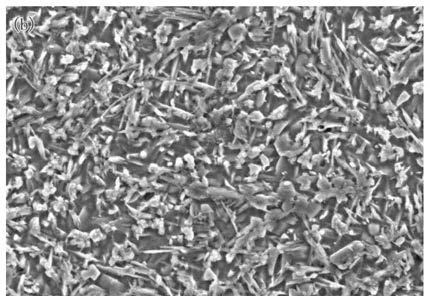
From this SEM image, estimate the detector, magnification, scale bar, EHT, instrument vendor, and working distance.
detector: SE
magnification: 2 K X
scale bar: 20000 nm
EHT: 15 kV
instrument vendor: Hitachi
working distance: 10.1 mm